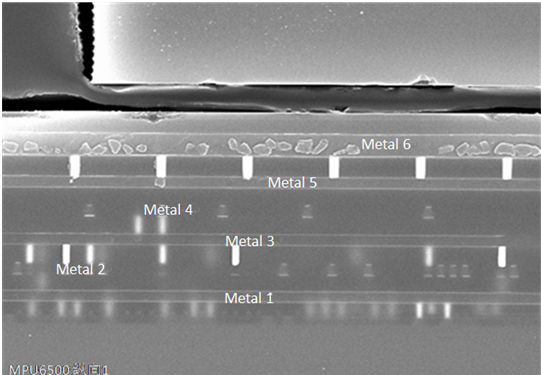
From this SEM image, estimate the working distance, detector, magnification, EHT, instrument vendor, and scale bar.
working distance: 11.6 mm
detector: SE(M)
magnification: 5 K X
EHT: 20 kV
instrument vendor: Hitachi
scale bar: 10000 nm